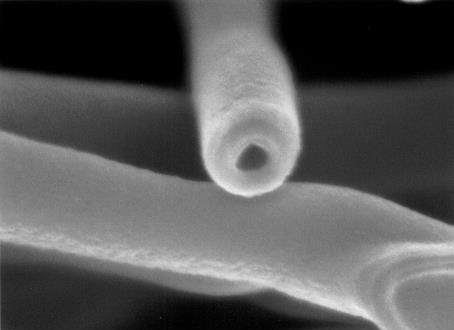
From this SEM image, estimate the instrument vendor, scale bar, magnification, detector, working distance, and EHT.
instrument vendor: JEOL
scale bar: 100 nm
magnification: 150 K X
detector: SEI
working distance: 2.9 mm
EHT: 3 kV